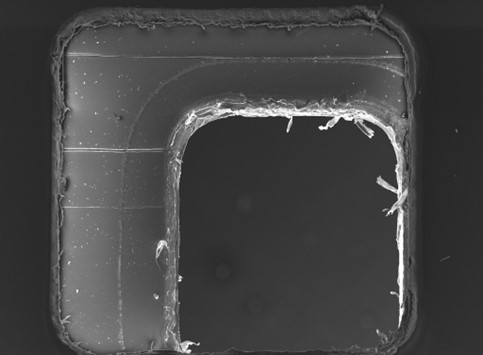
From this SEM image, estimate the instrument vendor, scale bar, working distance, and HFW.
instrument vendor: Tescan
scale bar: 500000 nm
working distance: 8.04 mm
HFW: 2740 µm